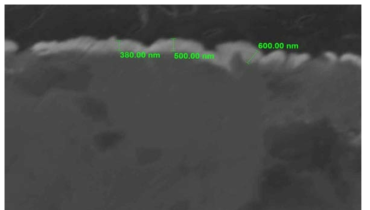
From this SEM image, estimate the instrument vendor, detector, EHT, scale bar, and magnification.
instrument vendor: JEOL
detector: SEI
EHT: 20 kV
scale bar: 1000 nm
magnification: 10 K X